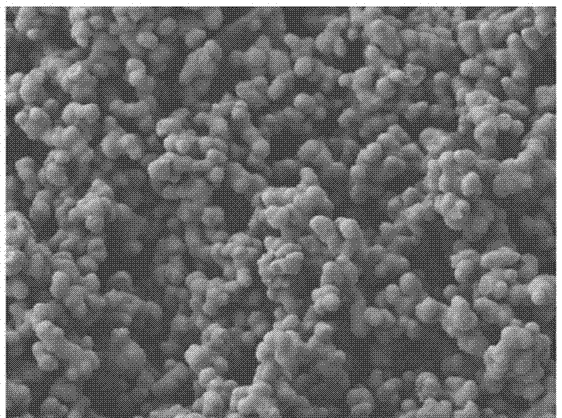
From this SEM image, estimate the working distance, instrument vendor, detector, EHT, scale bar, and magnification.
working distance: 9.6 mm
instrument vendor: JEOL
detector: LEI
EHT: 5 kV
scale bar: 1000 nm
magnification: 5 K X